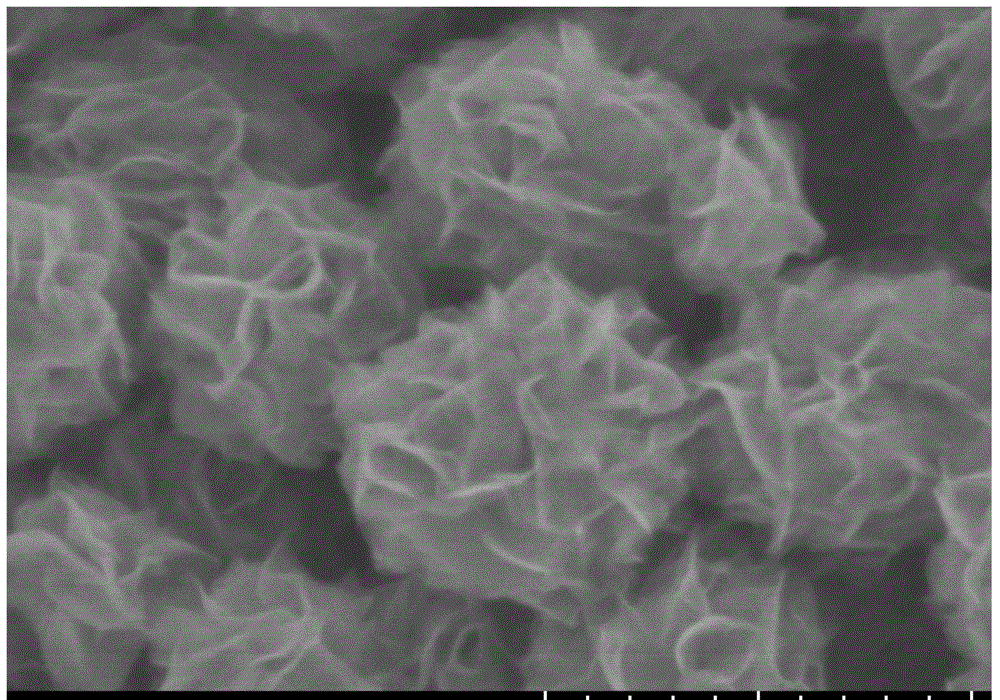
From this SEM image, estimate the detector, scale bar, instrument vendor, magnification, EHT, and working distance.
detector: SE(UL)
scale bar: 500 nm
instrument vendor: Hitachi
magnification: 110 K X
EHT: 2 kV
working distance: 8.8 mm